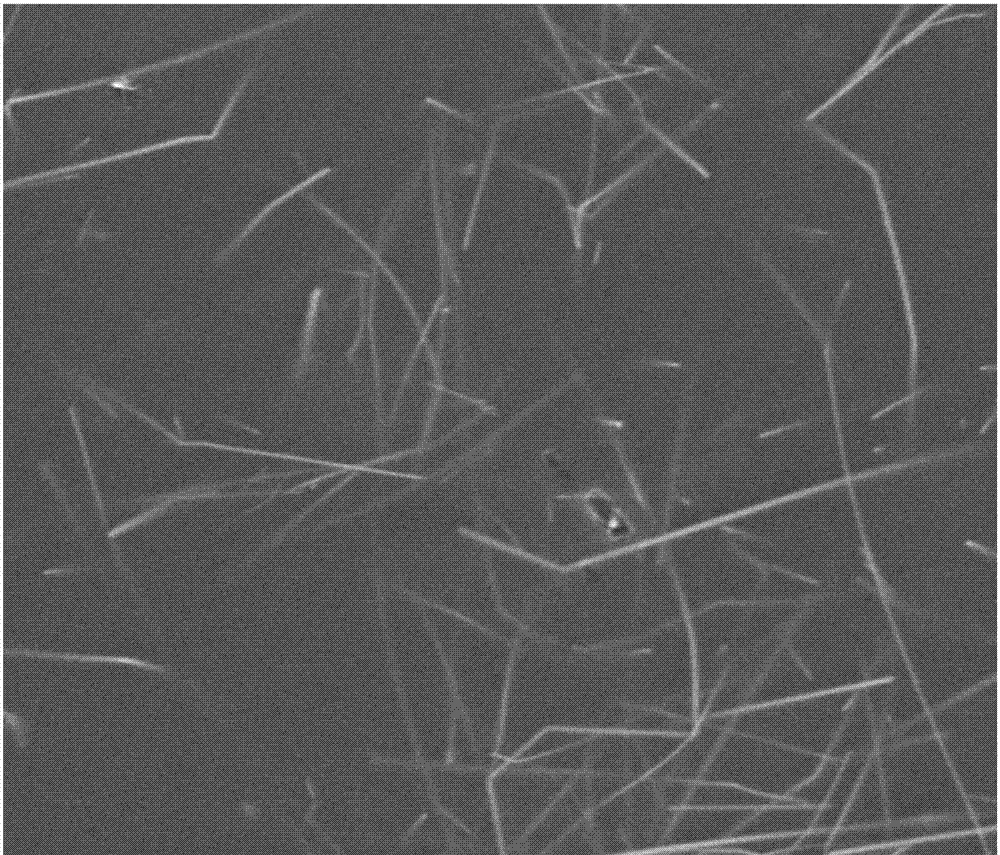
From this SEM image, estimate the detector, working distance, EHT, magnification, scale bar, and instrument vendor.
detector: ETD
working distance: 5 mm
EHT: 5 kV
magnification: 10 K X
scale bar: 4000 nm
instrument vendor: FEI